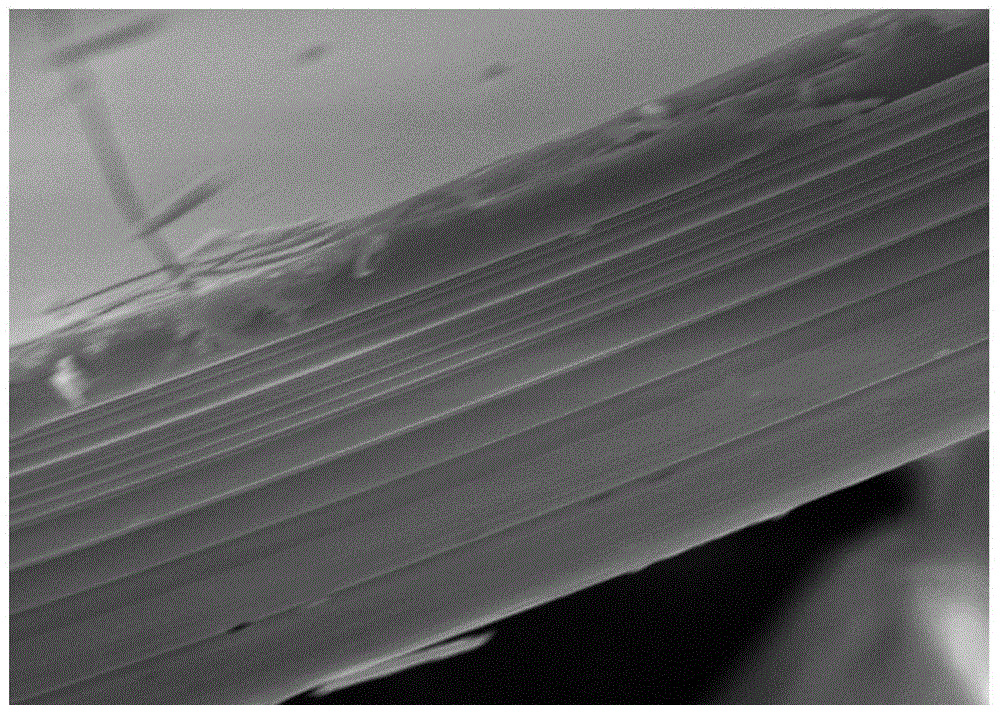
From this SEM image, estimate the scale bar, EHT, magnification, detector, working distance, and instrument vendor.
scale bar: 1000 nm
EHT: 10 kV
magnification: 10 K X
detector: InLens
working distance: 4.9 mm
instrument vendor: Zeiss